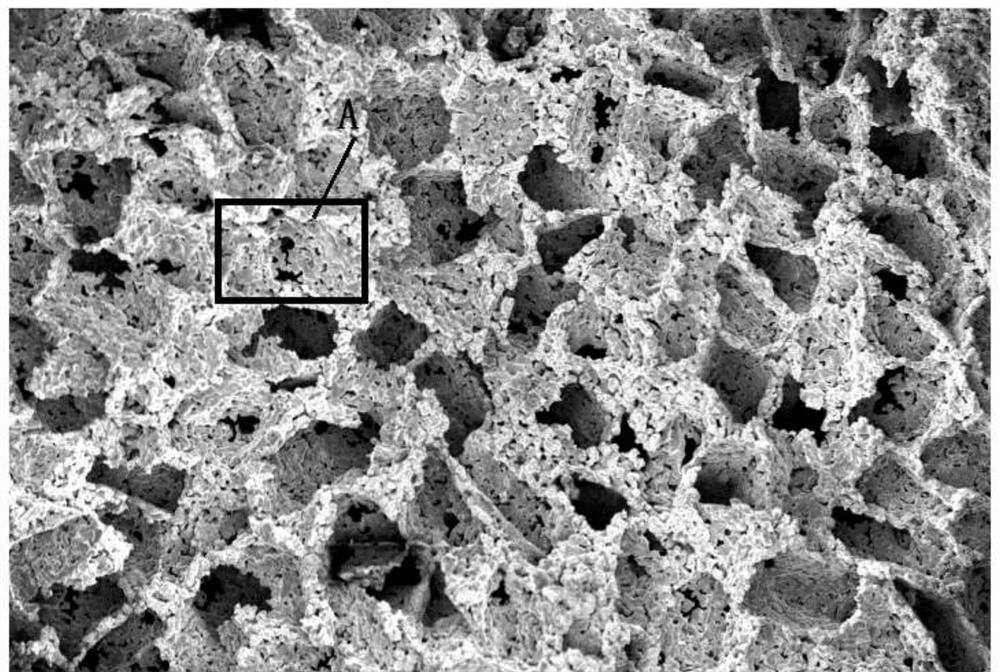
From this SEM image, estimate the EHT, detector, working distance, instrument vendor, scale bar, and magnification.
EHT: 20 kV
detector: InLens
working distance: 11.1 mm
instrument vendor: Zeiss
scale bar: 200000 nm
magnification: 0.08 K X